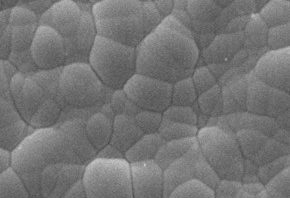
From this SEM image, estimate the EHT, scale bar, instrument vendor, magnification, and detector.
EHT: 10 kV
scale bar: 5000 nm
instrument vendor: JEOL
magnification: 5 K X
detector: SEI